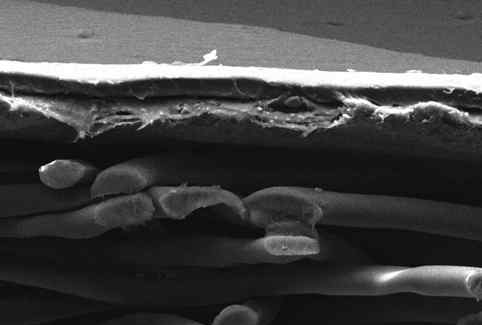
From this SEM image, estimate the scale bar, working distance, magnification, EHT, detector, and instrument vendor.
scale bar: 10000 nm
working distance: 8 mm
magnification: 0.5 K X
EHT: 15 kV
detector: SEI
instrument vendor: JEOL